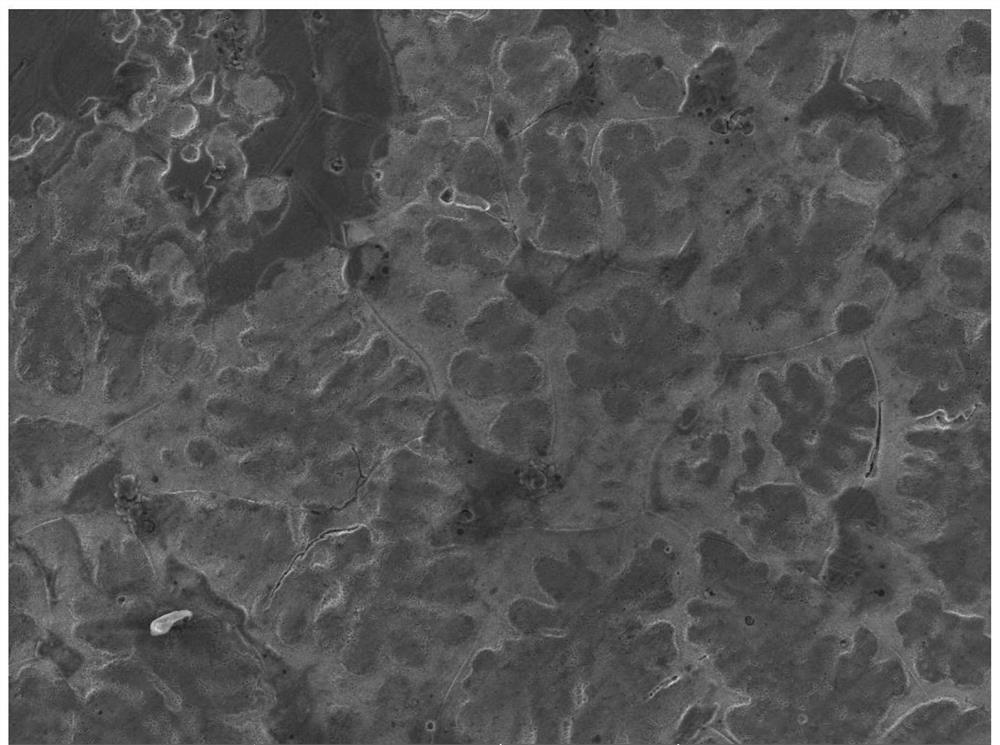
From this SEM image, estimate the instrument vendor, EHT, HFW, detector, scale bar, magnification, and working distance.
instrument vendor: Tescan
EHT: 20 kV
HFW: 554 µm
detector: SE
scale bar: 100000 nm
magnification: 0.5 K X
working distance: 9.92 mm